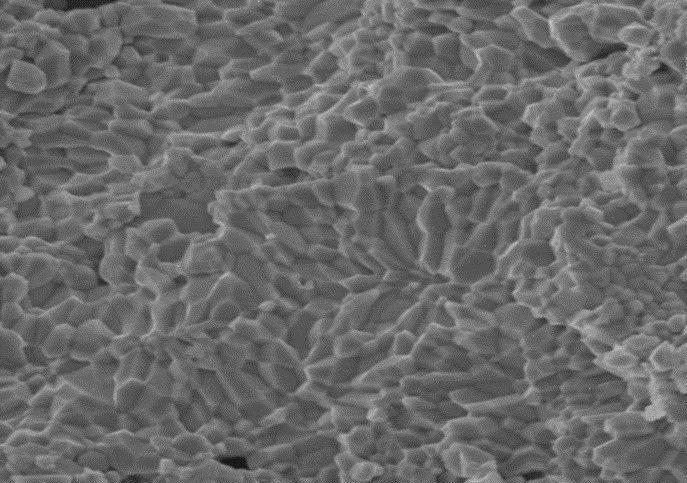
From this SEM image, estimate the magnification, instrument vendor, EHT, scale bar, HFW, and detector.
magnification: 20 K X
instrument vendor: Tescan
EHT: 10 kV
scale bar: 5000 nm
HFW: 17 µm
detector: SE